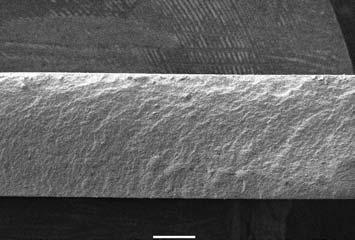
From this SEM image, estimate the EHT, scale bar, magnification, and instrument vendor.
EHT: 15 kV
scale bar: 500000 nm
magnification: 0.03 K X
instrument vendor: JEOL